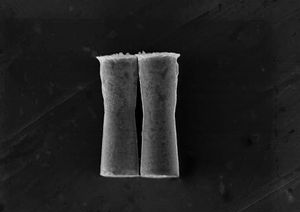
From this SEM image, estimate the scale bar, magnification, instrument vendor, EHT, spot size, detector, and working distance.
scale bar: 5000 nm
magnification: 3.498 K X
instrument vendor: FEI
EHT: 15 kV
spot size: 5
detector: SE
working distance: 10.8 mm